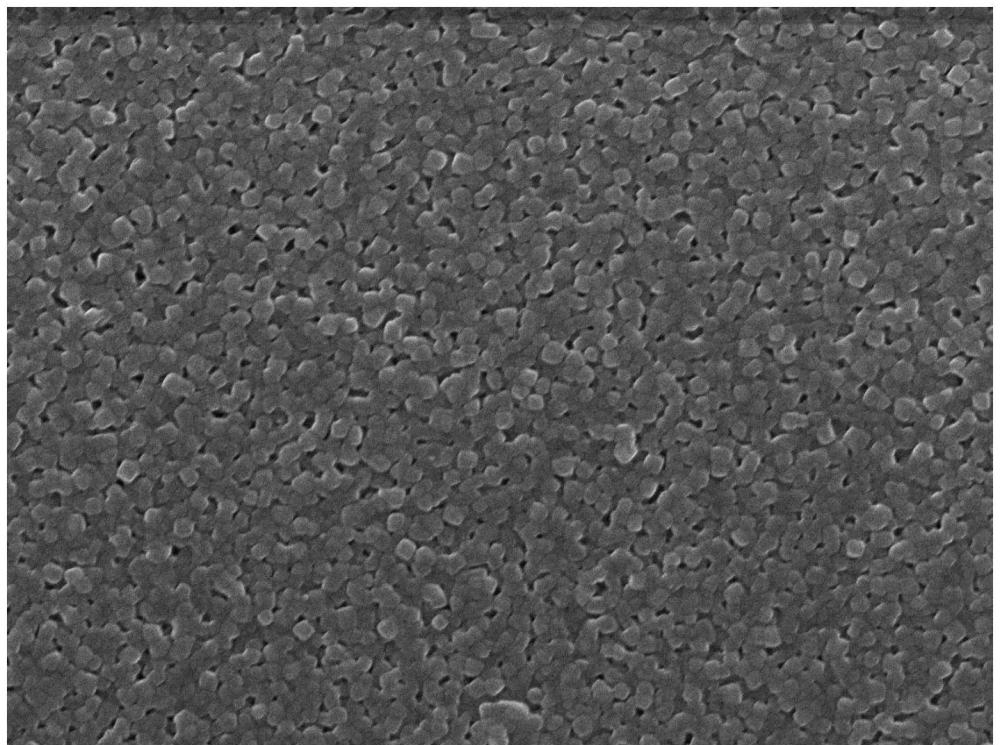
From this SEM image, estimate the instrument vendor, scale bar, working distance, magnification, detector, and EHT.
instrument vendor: JEOL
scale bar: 100 nm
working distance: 5.8 mm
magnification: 50 K X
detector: SEI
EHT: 10 kV